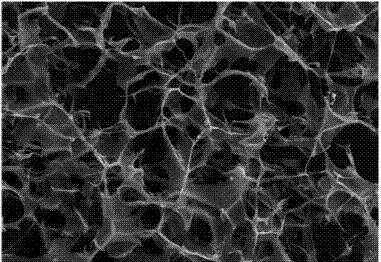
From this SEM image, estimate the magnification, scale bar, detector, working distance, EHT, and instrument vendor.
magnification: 0.25 K X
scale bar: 20000 nm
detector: SE1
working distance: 14.5 mm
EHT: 20 kV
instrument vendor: Zeiss